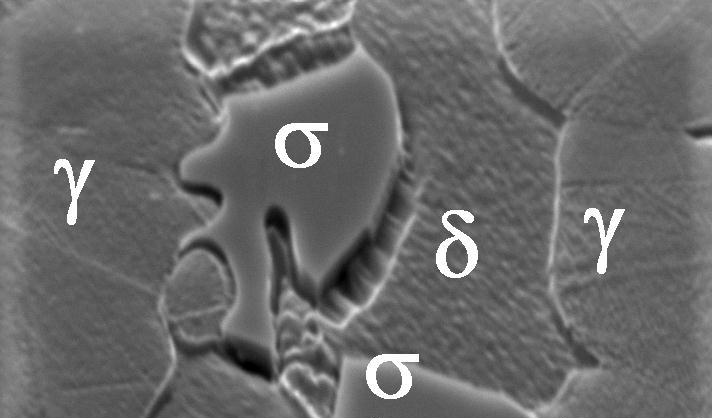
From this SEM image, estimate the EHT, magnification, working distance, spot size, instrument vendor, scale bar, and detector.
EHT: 25 kV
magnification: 6.226 K X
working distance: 15.1 mm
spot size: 5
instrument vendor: FEI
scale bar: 10000 nm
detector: SE